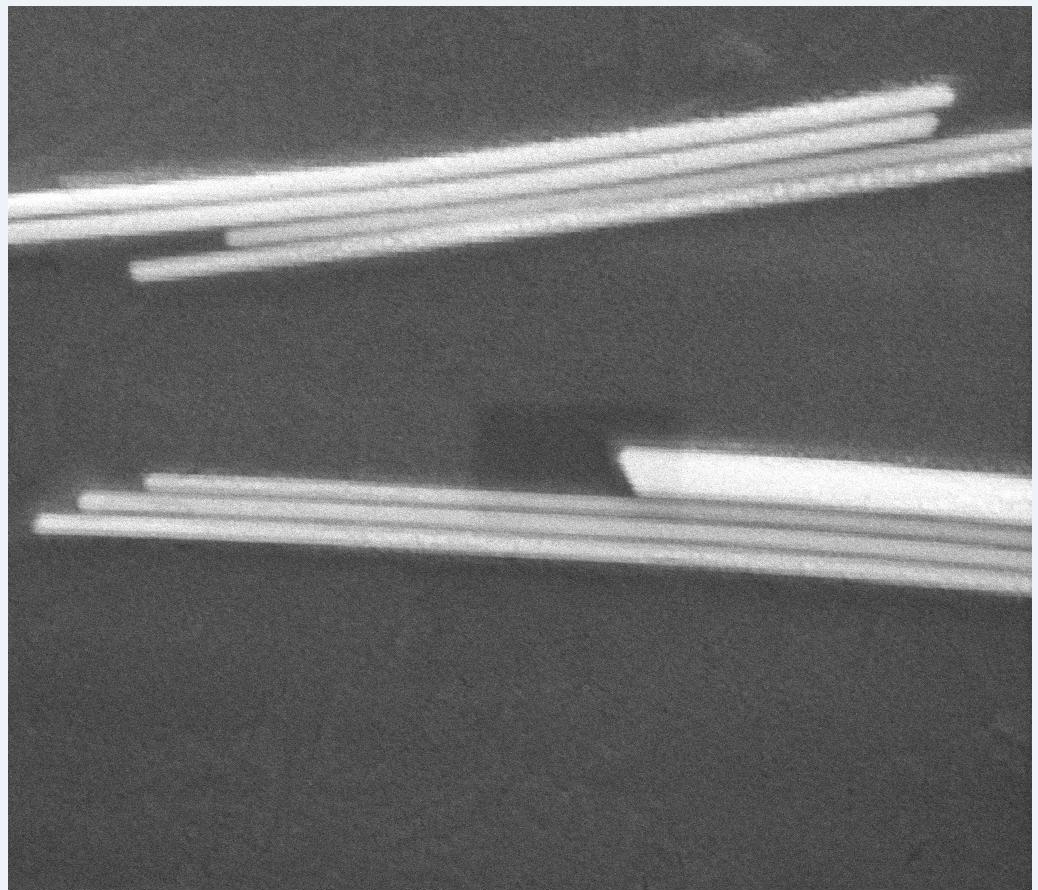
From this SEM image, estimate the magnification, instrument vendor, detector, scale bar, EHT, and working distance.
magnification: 50 K X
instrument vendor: FEI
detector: ETD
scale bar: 1000 nm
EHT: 20 kV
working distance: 10.9 mm